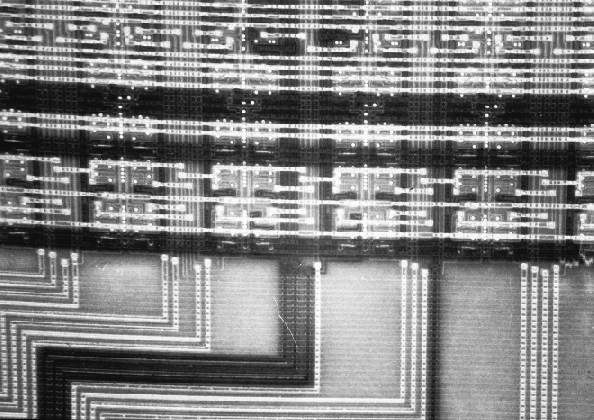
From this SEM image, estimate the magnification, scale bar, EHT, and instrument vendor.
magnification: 0.175 K X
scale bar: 57100 nm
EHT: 2 kV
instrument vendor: other